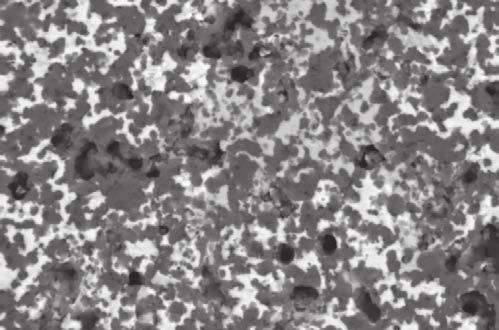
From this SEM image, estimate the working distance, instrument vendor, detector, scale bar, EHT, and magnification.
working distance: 16 mm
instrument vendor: Zeiss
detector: QBSD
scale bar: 10000 nm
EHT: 20 kV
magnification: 3 K X